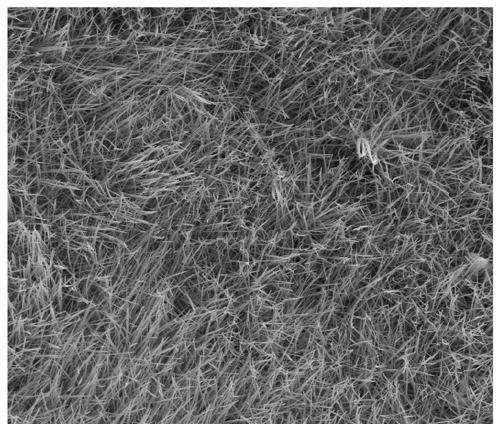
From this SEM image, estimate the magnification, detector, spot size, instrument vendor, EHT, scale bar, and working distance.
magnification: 8 K X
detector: ETD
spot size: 3.5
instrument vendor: FEI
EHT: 15 kV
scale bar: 10000 nm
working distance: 4.8 mm